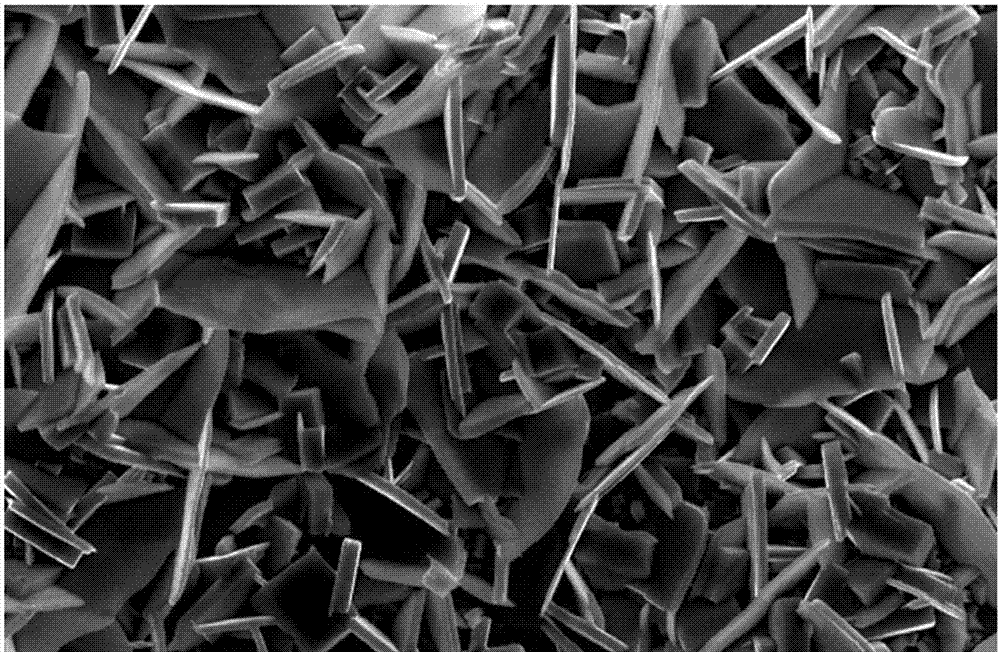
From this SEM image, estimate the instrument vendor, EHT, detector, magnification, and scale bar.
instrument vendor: JEOL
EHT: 20 kV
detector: SEI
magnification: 2 K X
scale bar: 10000 nm